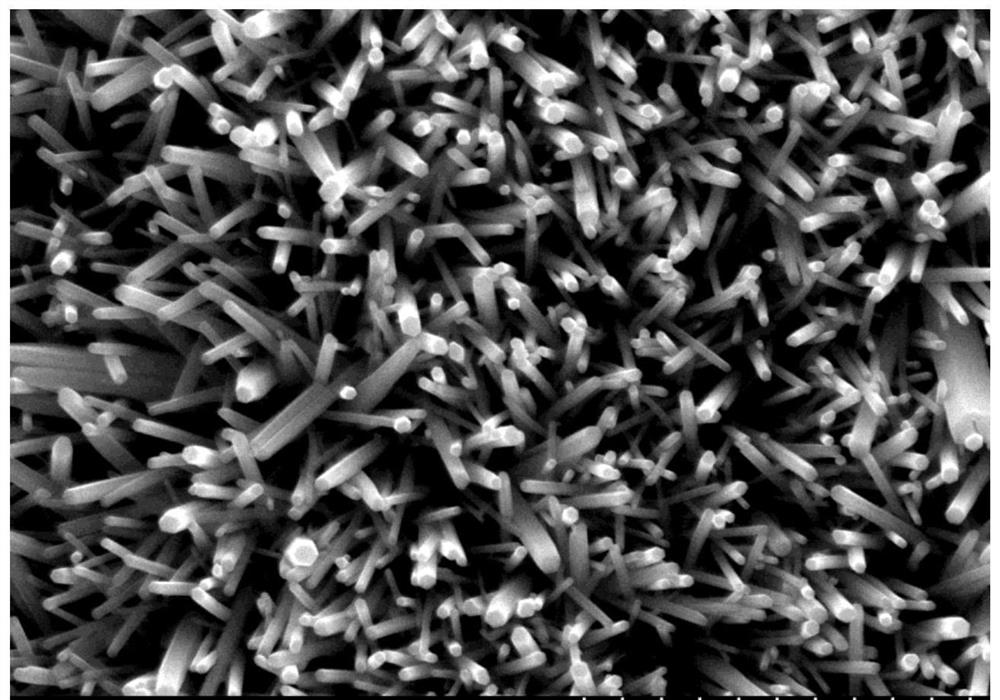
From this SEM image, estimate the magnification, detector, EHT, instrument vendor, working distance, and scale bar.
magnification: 25 K X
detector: SE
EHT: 20 kV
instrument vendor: Hitachi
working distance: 14.9 mm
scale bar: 2000 nm